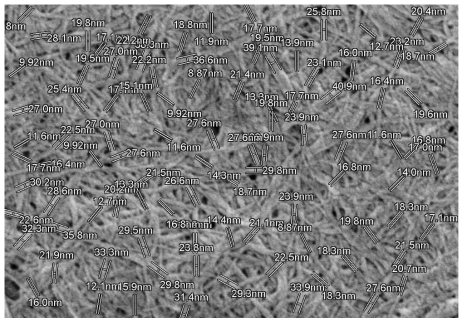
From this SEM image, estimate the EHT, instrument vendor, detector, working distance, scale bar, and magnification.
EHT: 1 kV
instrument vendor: Hitachi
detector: SE(U)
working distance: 9.4 mm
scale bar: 1000 nm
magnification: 50 K X